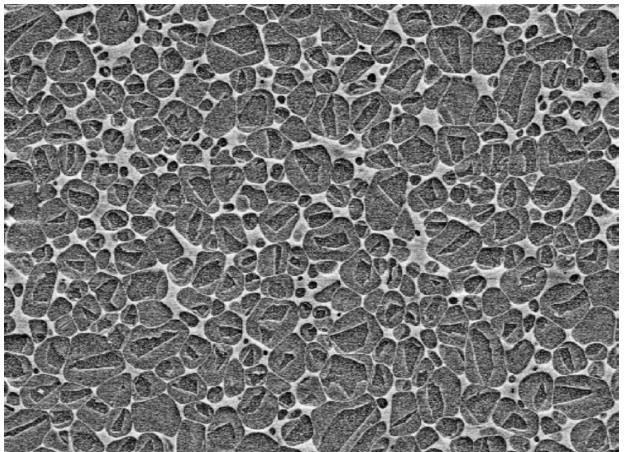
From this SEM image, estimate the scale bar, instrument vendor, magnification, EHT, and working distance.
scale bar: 2000 nm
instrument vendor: Zeiss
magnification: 10 K X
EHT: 5 kV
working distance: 3 mm